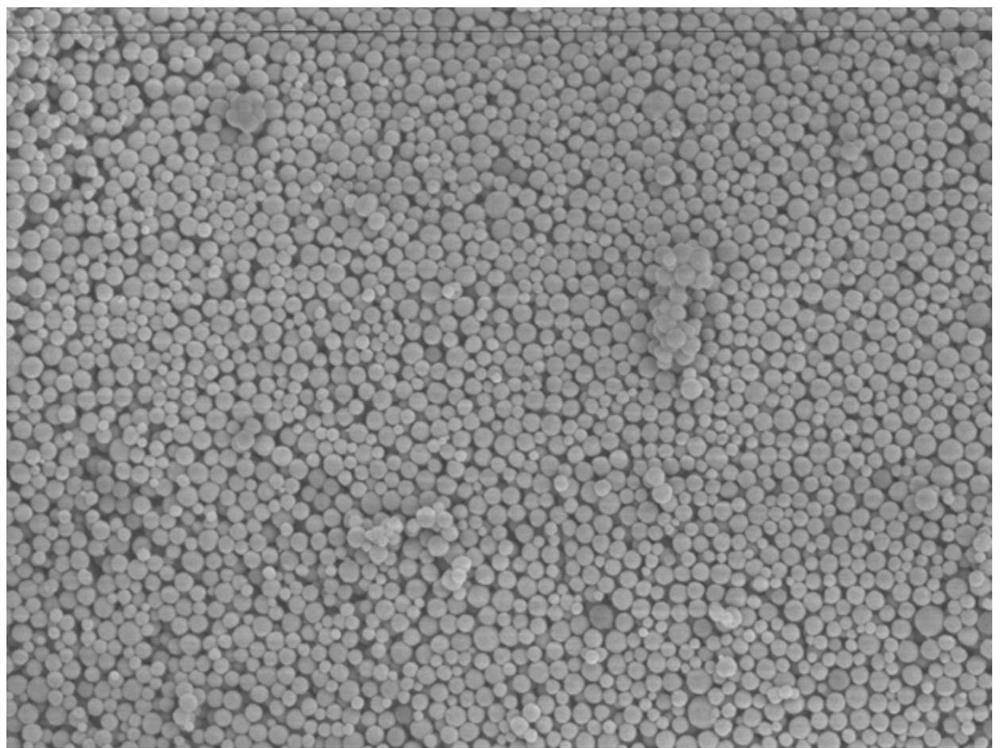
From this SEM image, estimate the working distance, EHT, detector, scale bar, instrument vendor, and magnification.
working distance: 37.2 mm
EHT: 3 kV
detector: SEI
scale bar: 1000 nm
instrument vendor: JEOL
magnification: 10 K X